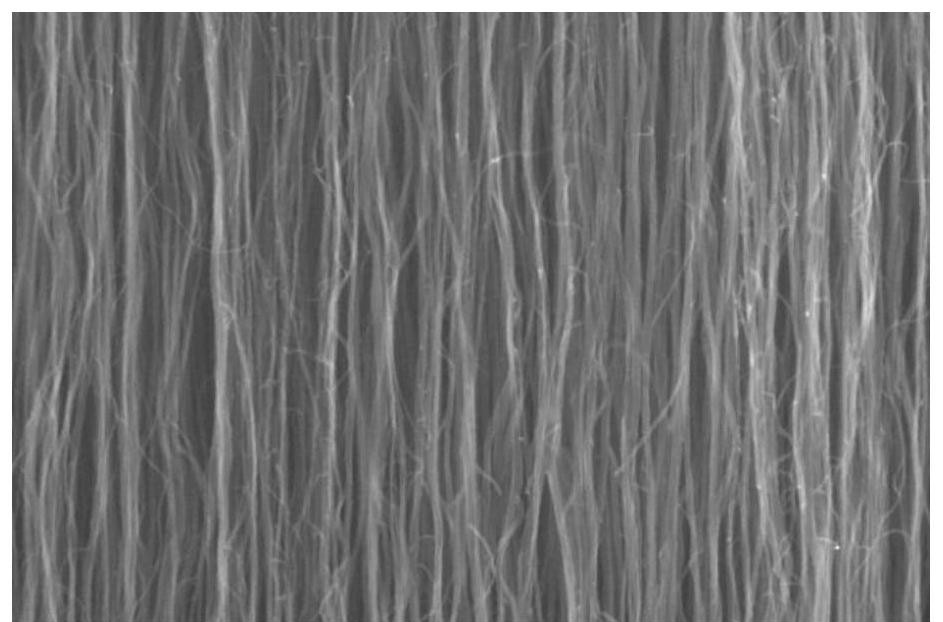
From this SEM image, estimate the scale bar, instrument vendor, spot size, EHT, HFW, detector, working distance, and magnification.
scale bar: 1000 nm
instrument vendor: FEI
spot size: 3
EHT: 20 kV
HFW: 4.14 µm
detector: ETD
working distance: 10 mm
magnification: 100 K X